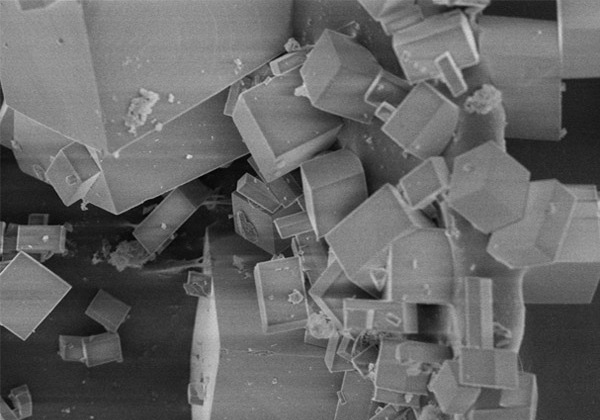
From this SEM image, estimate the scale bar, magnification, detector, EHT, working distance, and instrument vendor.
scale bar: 20000 nm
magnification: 2.5 K X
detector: SE(M)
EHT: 3 kV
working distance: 9.3 mm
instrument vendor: Hitachi